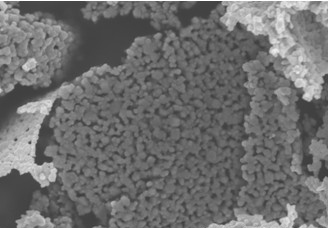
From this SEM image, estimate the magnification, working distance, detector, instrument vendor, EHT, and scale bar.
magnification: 25 K X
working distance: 9.4 mm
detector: SE(M)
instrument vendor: Hitachi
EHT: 5 kV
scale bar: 2000 nm